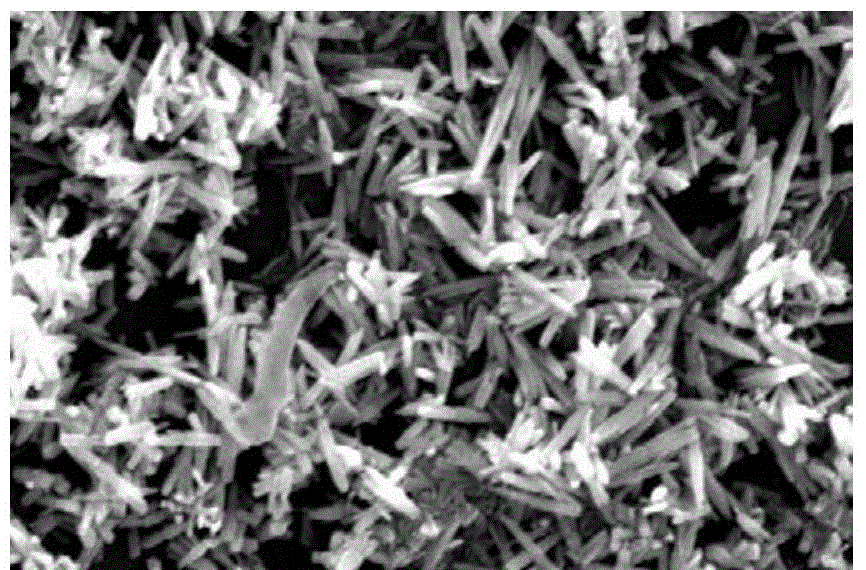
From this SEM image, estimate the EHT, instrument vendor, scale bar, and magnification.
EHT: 20 kV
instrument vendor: JEOL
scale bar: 1000 nm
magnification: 10 K X